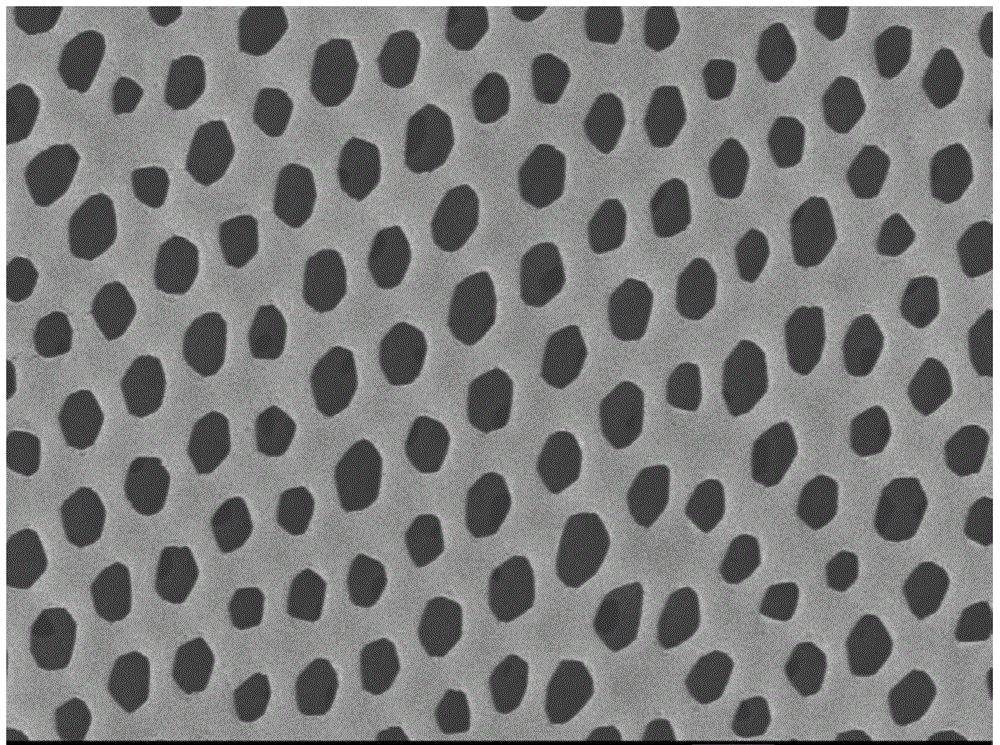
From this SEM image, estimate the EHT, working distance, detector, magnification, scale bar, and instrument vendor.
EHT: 3 kV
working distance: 7.6 mm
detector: LEI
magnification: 10 K X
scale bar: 1000 nm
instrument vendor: JEOL